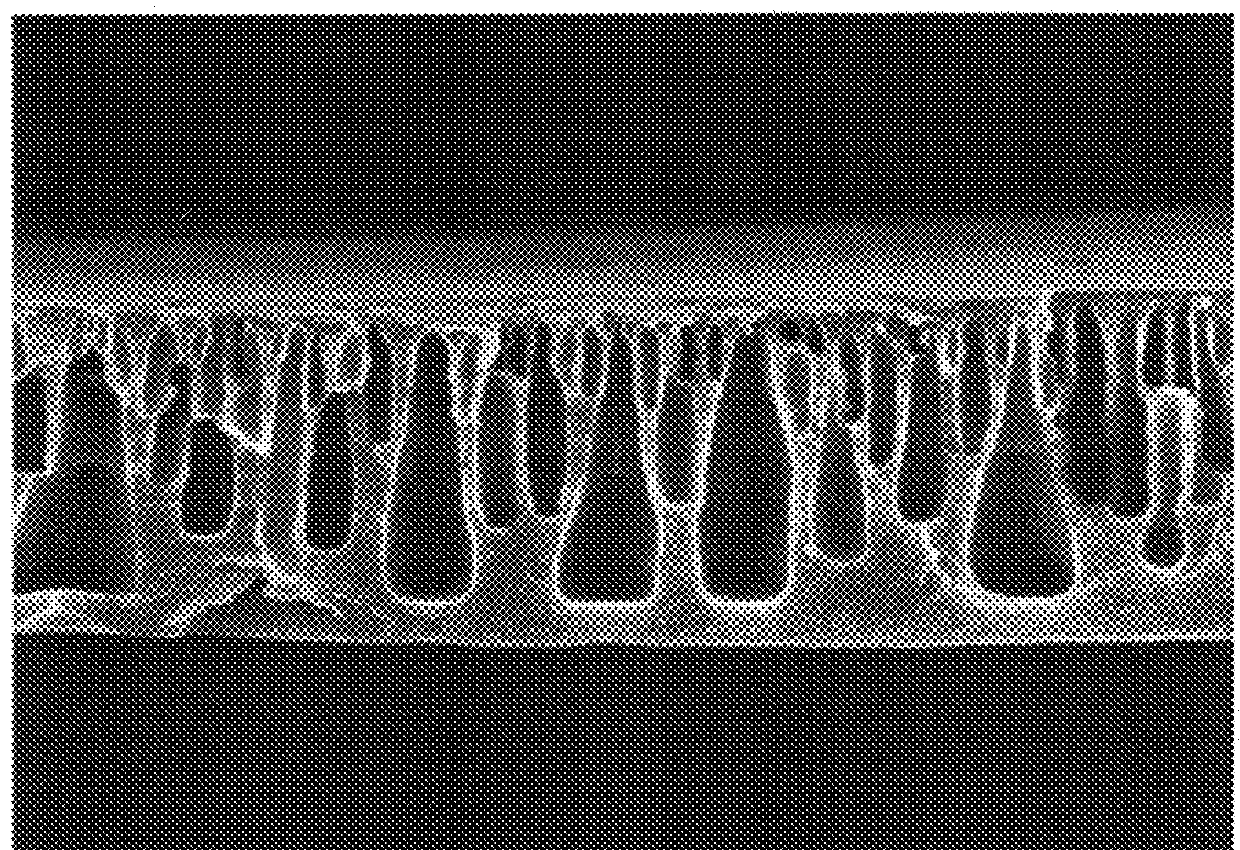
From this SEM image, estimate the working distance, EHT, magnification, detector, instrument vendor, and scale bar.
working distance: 6.8 mm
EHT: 15 kV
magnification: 0.7 K X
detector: SE(M)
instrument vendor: Hitachi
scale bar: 50000 nm